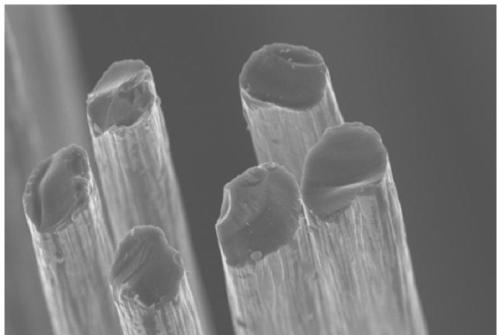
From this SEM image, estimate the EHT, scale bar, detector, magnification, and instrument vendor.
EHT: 8 kV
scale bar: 10000 nm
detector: SE(M)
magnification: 3 K X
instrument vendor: Hitachi